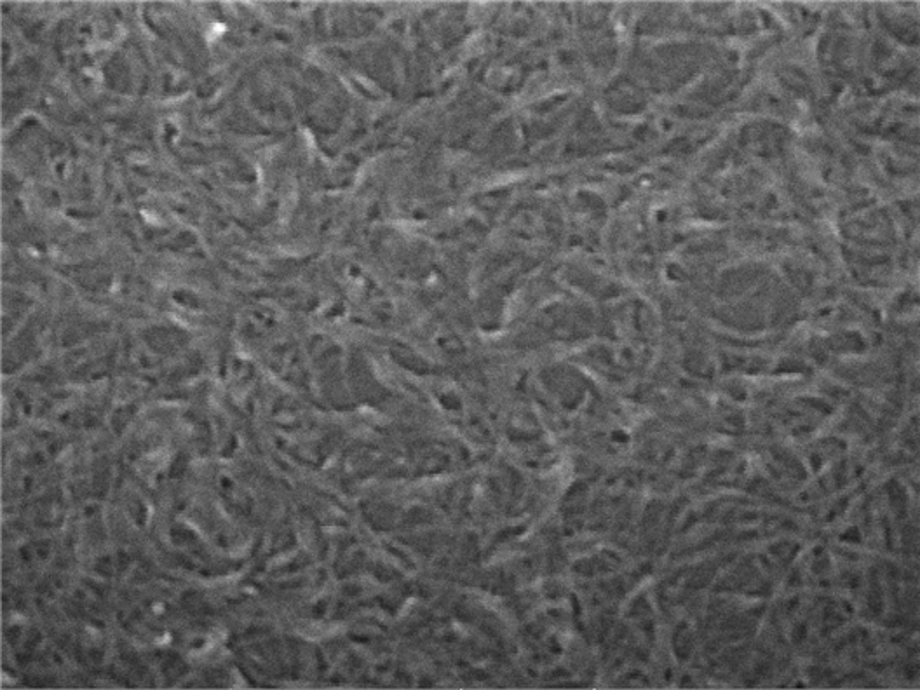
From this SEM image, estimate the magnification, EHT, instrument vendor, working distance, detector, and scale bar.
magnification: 200 K X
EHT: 15 kV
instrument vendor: Tescan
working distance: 11.76 mm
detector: InBeam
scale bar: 200 nm